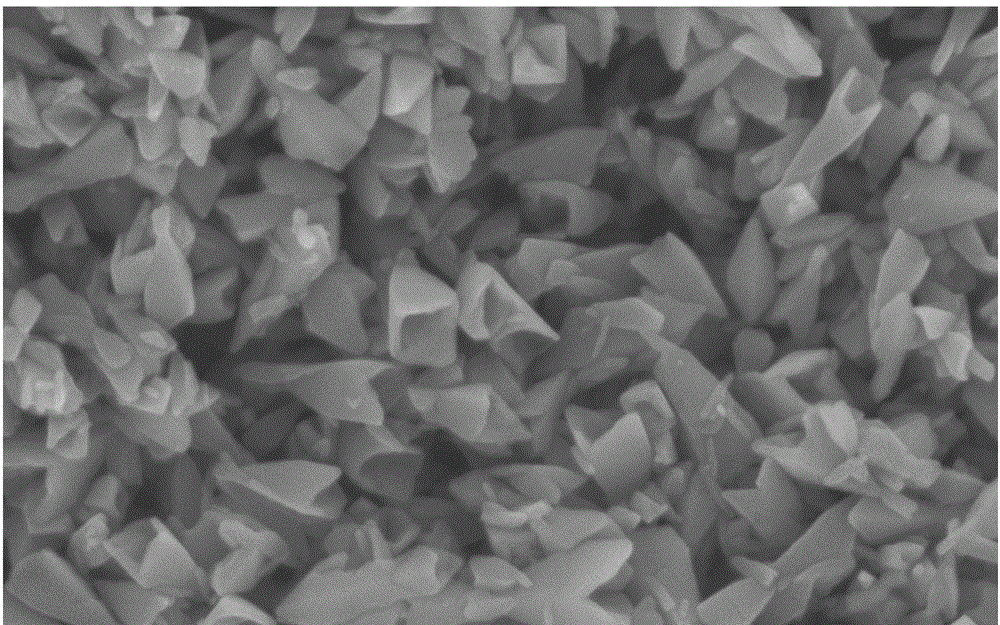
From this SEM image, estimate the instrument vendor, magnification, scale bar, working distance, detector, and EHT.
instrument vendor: Hitachi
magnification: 60 K X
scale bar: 500 nm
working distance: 8.7 mm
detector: SE(M)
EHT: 5 kV